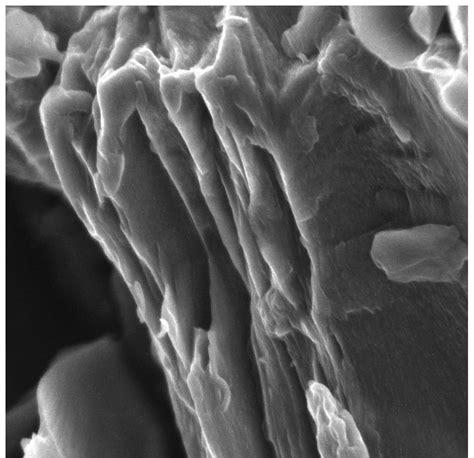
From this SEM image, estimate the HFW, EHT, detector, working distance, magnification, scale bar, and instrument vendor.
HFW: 5.19 µm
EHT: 5 kV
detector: In-Beam SE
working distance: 4.96 mm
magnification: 40 K X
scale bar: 1000 nm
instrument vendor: Tescan